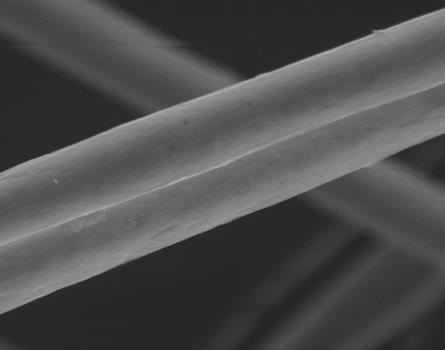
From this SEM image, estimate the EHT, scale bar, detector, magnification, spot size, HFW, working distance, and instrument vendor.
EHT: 15 kV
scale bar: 20000 nm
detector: LVD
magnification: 6 K X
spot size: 4.5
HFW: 49.7 µm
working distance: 5.1 mm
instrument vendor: FEI